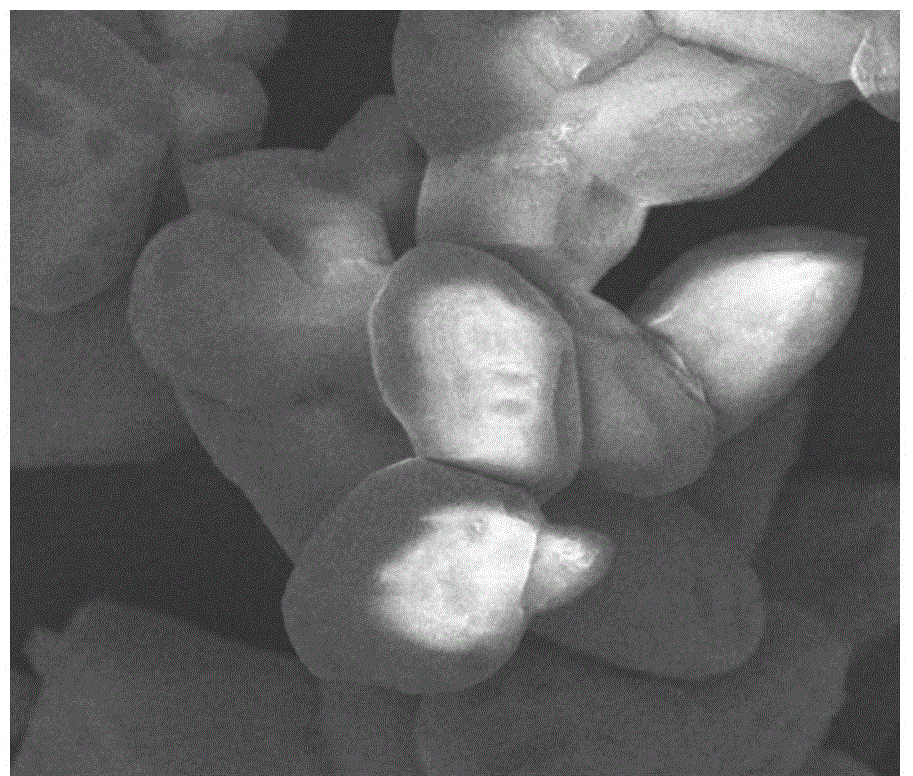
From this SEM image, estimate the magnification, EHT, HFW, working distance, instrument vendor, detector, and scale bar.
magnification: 15 K X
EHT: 10 kV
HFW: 16 µm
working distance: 5.1 mm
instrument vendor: FEI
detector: TLD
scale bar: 5000 nm